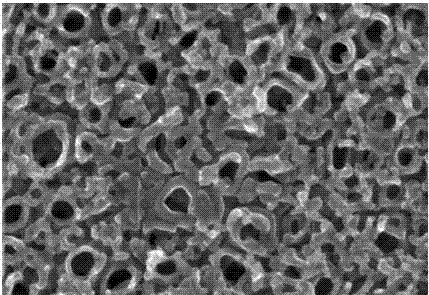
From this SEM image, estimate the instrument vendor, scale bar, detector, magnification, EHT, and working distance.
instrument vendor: Hitachi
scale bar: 500 nm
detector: SE(M)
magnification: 100 K X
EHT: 15 kV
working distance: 10.7 mm